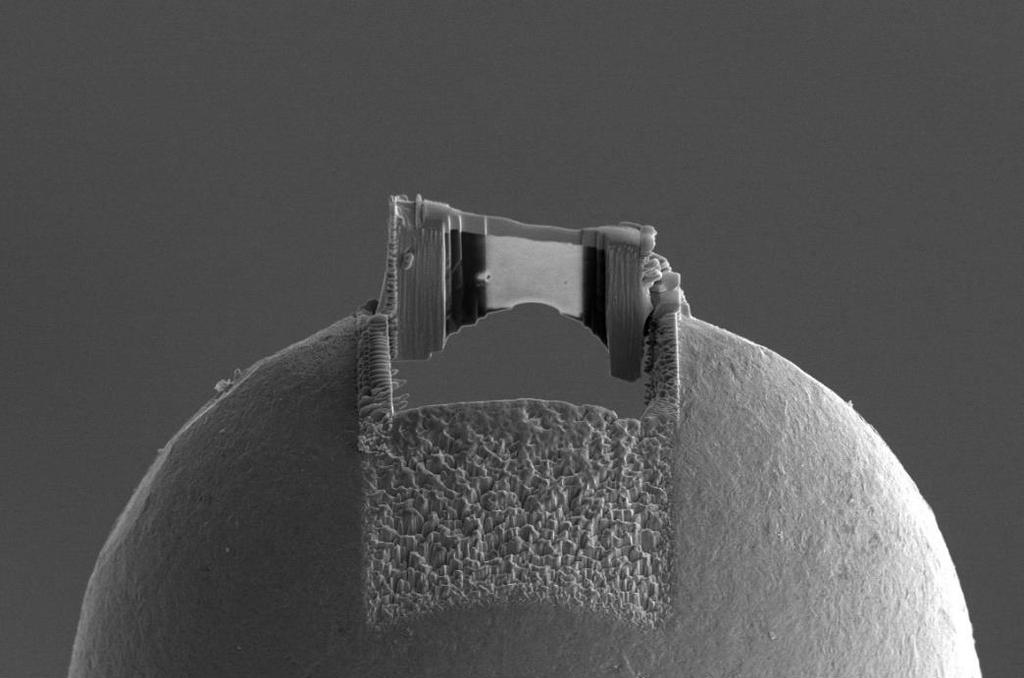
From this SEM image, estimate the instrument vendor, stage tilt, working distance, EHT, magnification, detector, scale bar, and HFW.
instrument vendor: FEI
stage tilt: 52°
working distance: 3.9 mm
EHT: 5 kV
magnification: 2.5 K X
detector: ETD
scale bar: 10000 nm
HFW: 82.9 µm